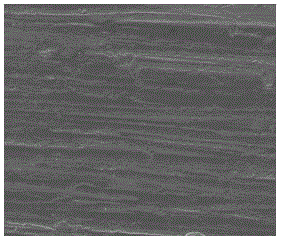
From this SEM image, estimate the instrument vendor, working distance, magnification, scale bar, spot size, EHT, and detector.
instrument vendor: FEI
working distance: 7.7 mm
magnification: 1 K X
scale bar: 50000 nm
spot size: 3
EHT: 10 kV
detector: ETD